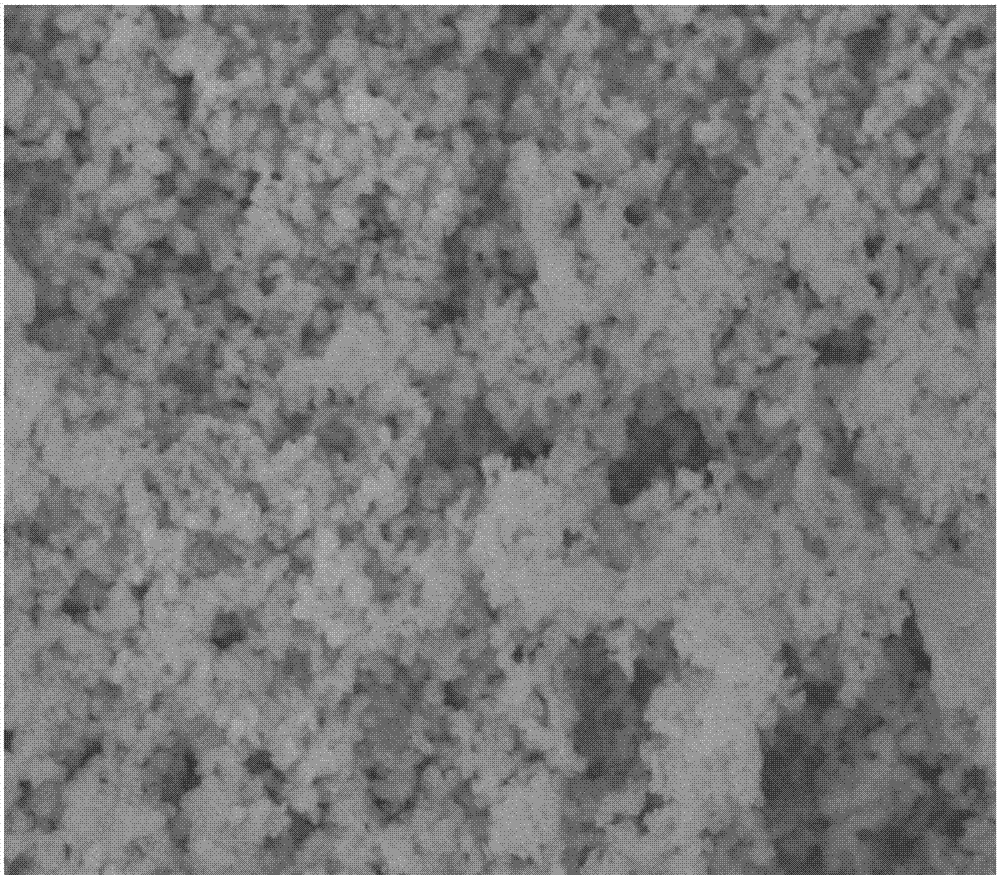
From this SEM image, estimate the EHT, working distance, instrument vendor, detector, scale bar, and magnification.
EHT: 10 kV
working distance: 8.7 mm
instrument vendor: JEOL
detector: SEI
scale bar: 1000 nm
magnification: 20 K X